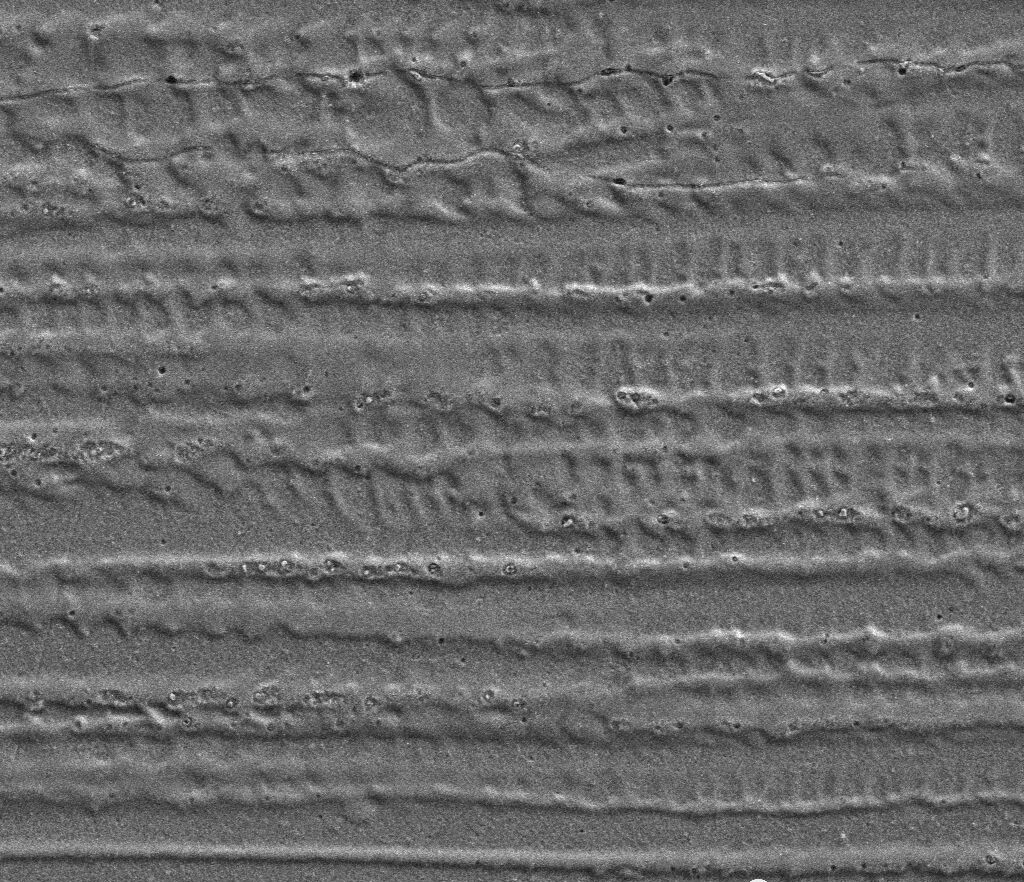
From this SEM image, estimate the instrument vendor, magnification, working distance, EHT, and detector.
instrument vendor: FEI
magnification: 1 K X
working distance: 8 mm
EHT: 20 kV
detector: ETD